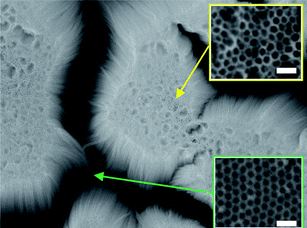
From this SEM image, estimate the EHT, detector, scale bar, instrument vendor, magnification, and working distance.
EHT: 20 kV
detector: SEI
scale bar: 1000 nm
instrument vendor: JEOL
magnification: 25 K X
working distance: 22.6 mm